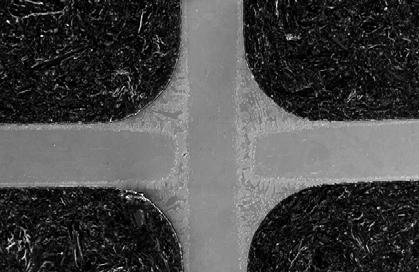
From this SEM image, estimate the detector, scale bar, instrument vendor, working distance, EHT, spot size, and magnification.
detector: SE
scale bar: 500000 nm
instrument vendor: FEI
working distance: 10.3 mm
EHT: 20 kV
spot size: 5.1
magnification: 0.1 K X